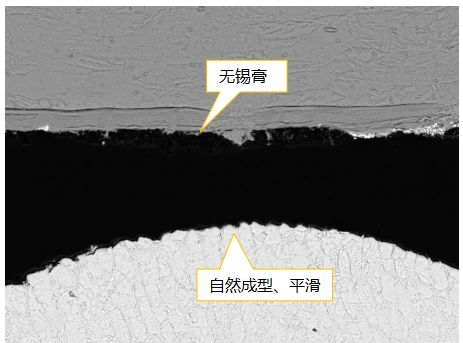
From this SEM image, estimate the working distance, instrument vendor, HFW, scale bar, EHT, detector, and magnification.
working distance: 14.9 mm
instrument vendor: Tescan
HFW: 154 µm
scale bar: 20000 nm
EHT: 20 kV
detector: BSE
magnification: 1.79 K X